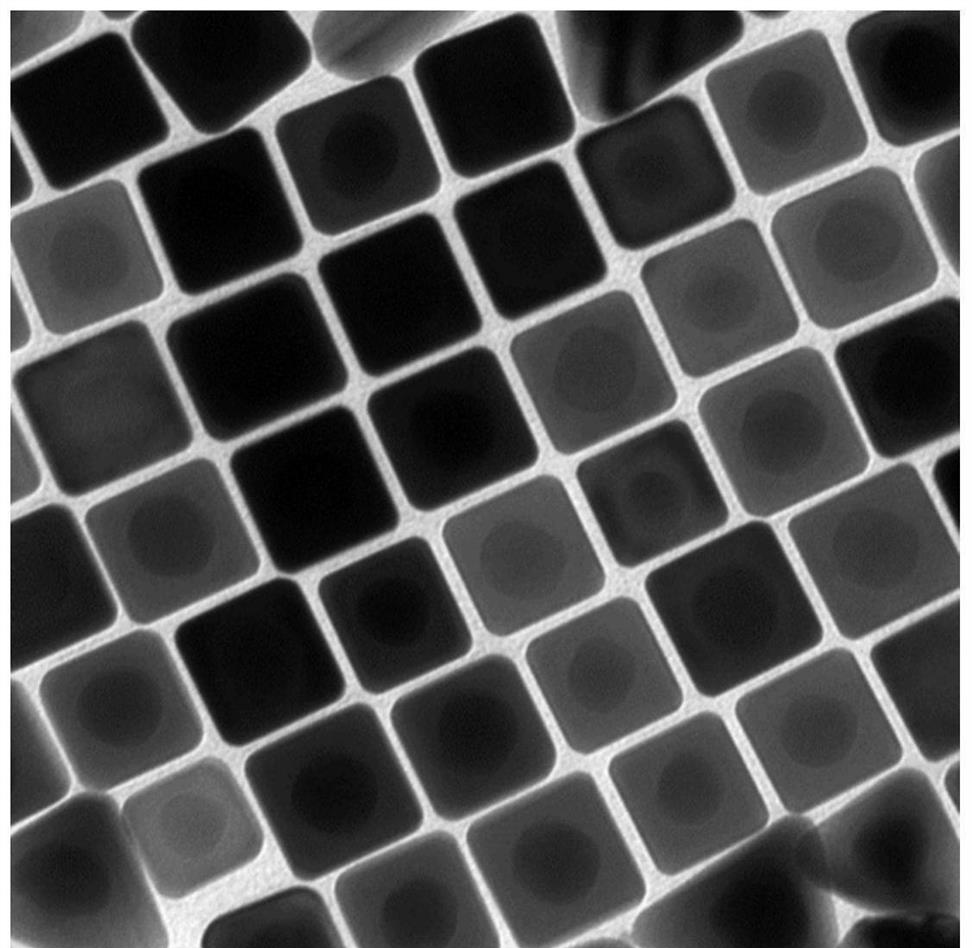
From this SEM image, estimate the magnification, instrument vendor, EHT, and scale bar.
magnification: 60 K X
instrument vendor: Hitachi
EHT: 100 kV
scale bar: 100 nm